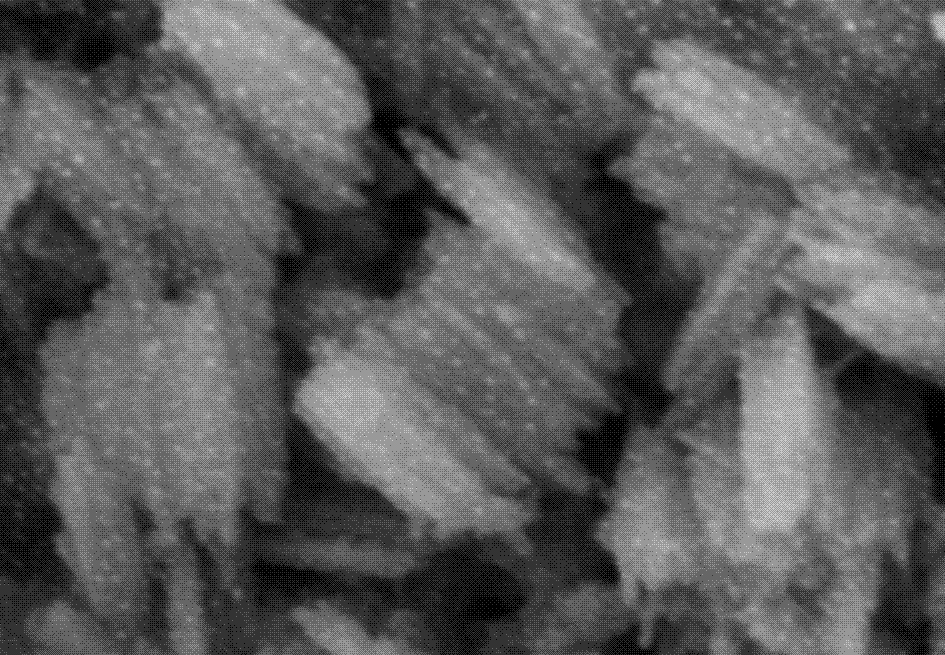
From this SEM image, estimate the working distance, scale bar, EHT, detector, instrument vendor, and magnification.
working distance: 13.6 mm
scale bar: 500 nm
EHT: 5 kV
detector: SE(M)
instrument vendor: Hitachi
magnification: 100 K X